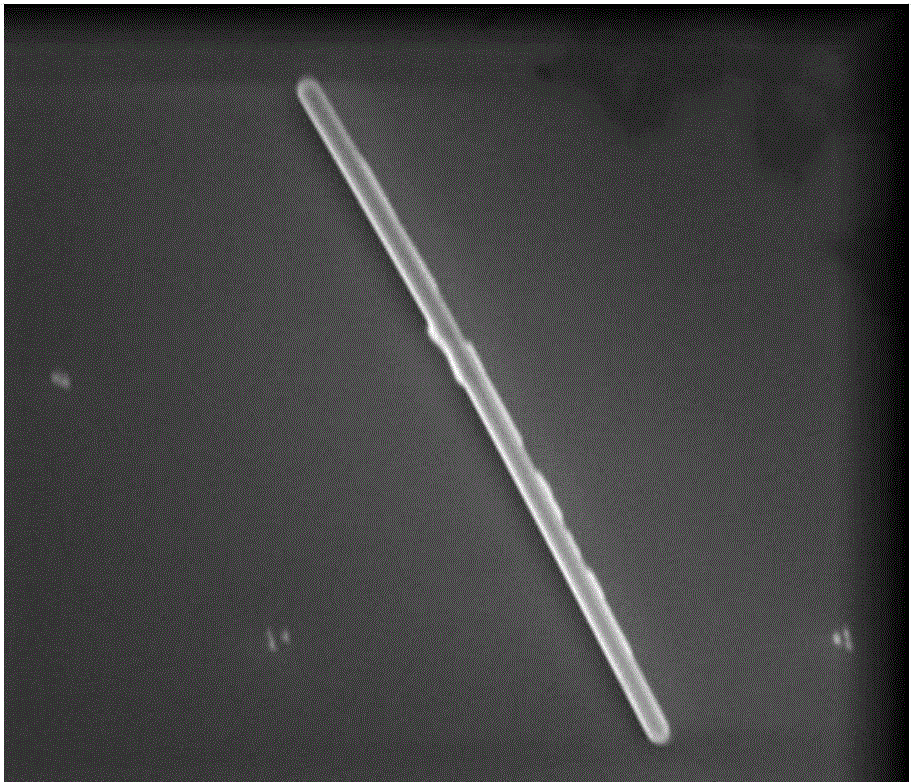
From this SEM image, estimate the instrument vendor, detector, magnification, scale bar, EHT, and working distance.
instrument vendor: FEI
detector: ETD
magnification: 20 K X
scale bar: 2000 nm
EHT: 10 kV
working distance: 9 mm